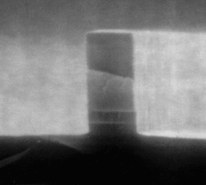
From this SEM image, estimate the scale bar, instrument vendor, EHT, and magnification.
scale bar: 50000 nm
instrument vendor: JEOL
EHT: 10 kV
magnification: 20 K X